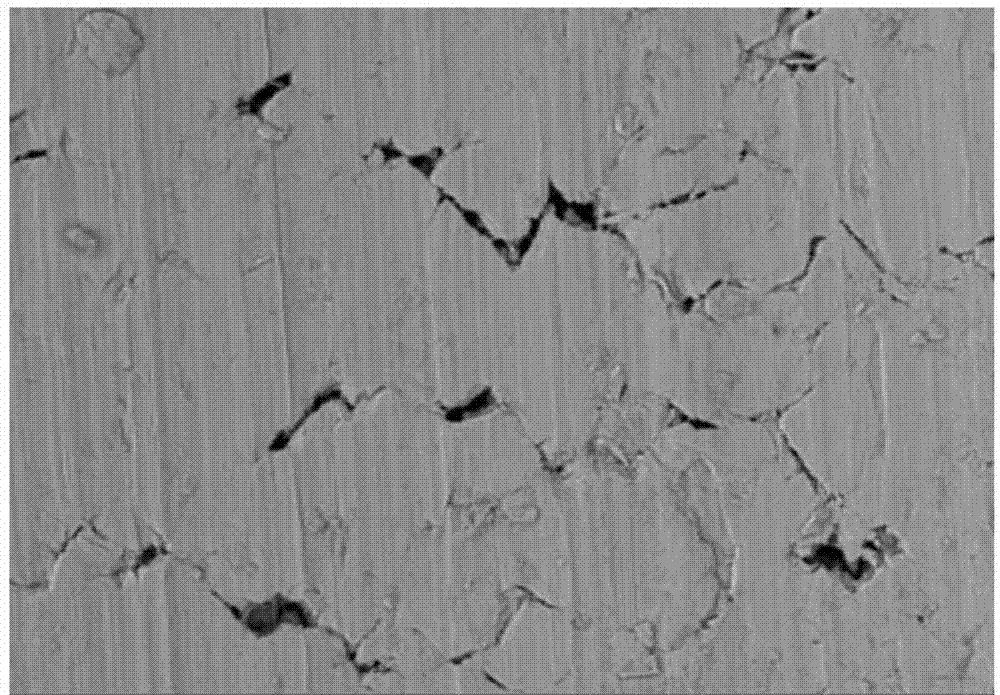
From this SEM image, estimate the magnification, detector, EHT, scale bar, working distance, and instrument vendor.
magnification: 1 K X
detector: BSE3D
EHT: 15 kV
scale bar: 50000 nm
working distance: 9.2 mm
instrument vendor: Hitachi